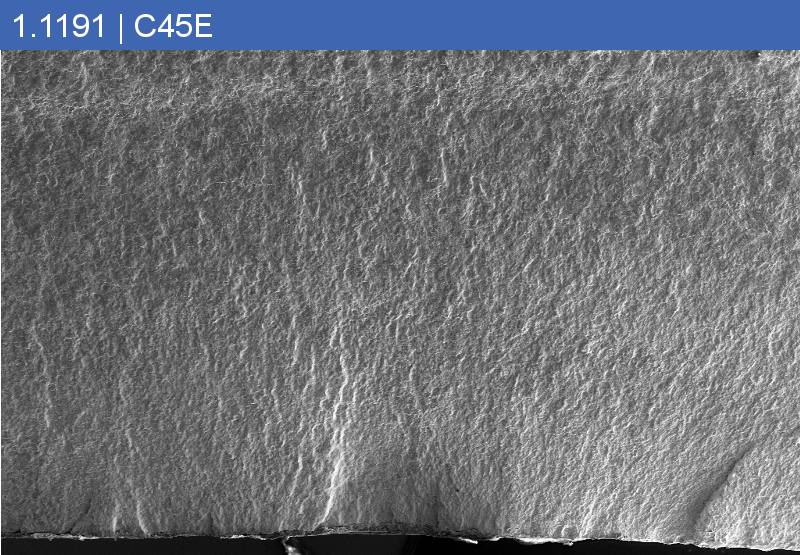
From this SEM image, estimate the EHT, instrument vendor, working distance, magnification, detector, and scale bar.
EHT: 15 kV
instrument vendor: Zeiss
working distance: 14.4 mm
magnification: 0.02 K X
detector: SE2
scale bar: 200000 nm